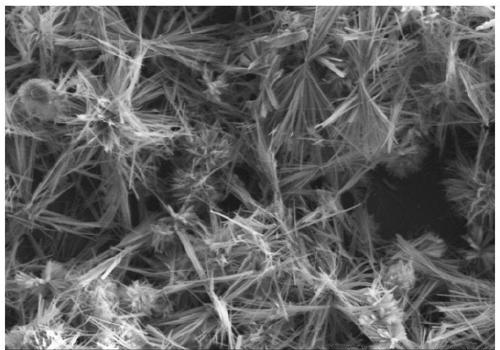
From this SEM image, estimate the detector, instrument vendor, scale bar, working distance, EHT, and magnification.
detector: SE(M)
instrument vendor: Hitachi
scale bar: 200000 nm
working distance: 15.2 mm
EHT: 15 kV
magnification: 0.2 K X